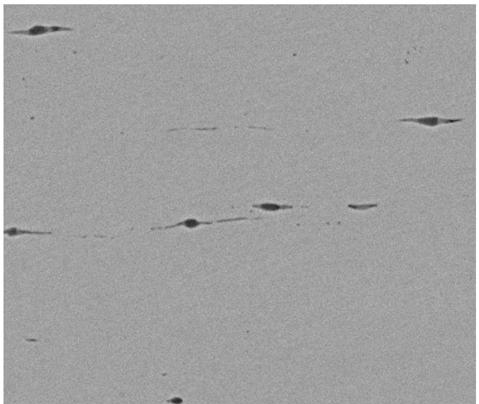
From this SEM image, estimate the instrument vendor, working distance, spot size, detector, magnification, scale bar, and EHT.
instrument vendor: FEI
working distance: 11.5 mm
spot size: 5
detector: SSD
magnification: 2 K X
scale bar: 20000 nm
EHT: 20 kV